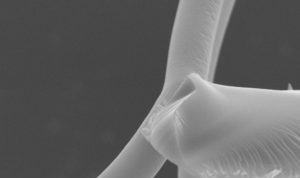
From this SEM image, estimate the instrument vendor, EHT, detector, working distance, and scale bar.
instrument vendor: Zeiss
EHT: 20 kV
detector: SE1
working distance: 15 mm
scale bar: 2000 nm